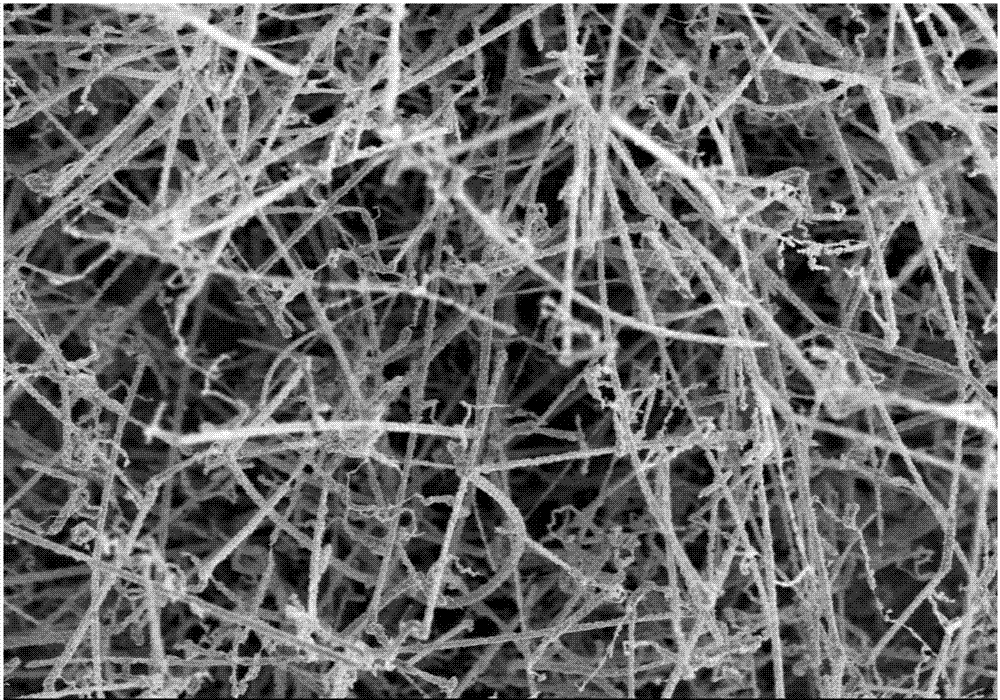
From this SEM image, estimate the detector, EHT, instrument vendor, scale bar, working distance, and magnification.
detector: SE(M)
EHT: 5 kV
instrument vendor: Hitachi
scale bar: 10000 nm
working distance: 8.7 mm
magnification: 4 K X